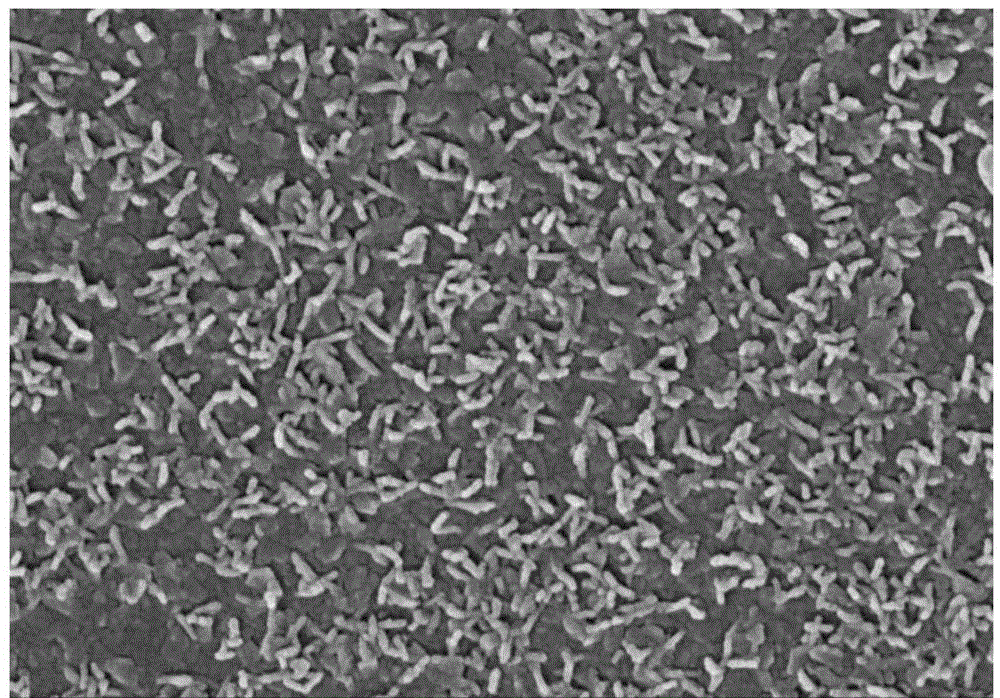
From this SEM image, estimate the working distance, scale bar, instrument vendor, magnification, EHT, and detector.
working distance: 8.2 mm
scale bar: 1000 nm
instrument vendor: Hitachi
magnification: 30 K X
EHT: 15 kV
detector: SE(M)